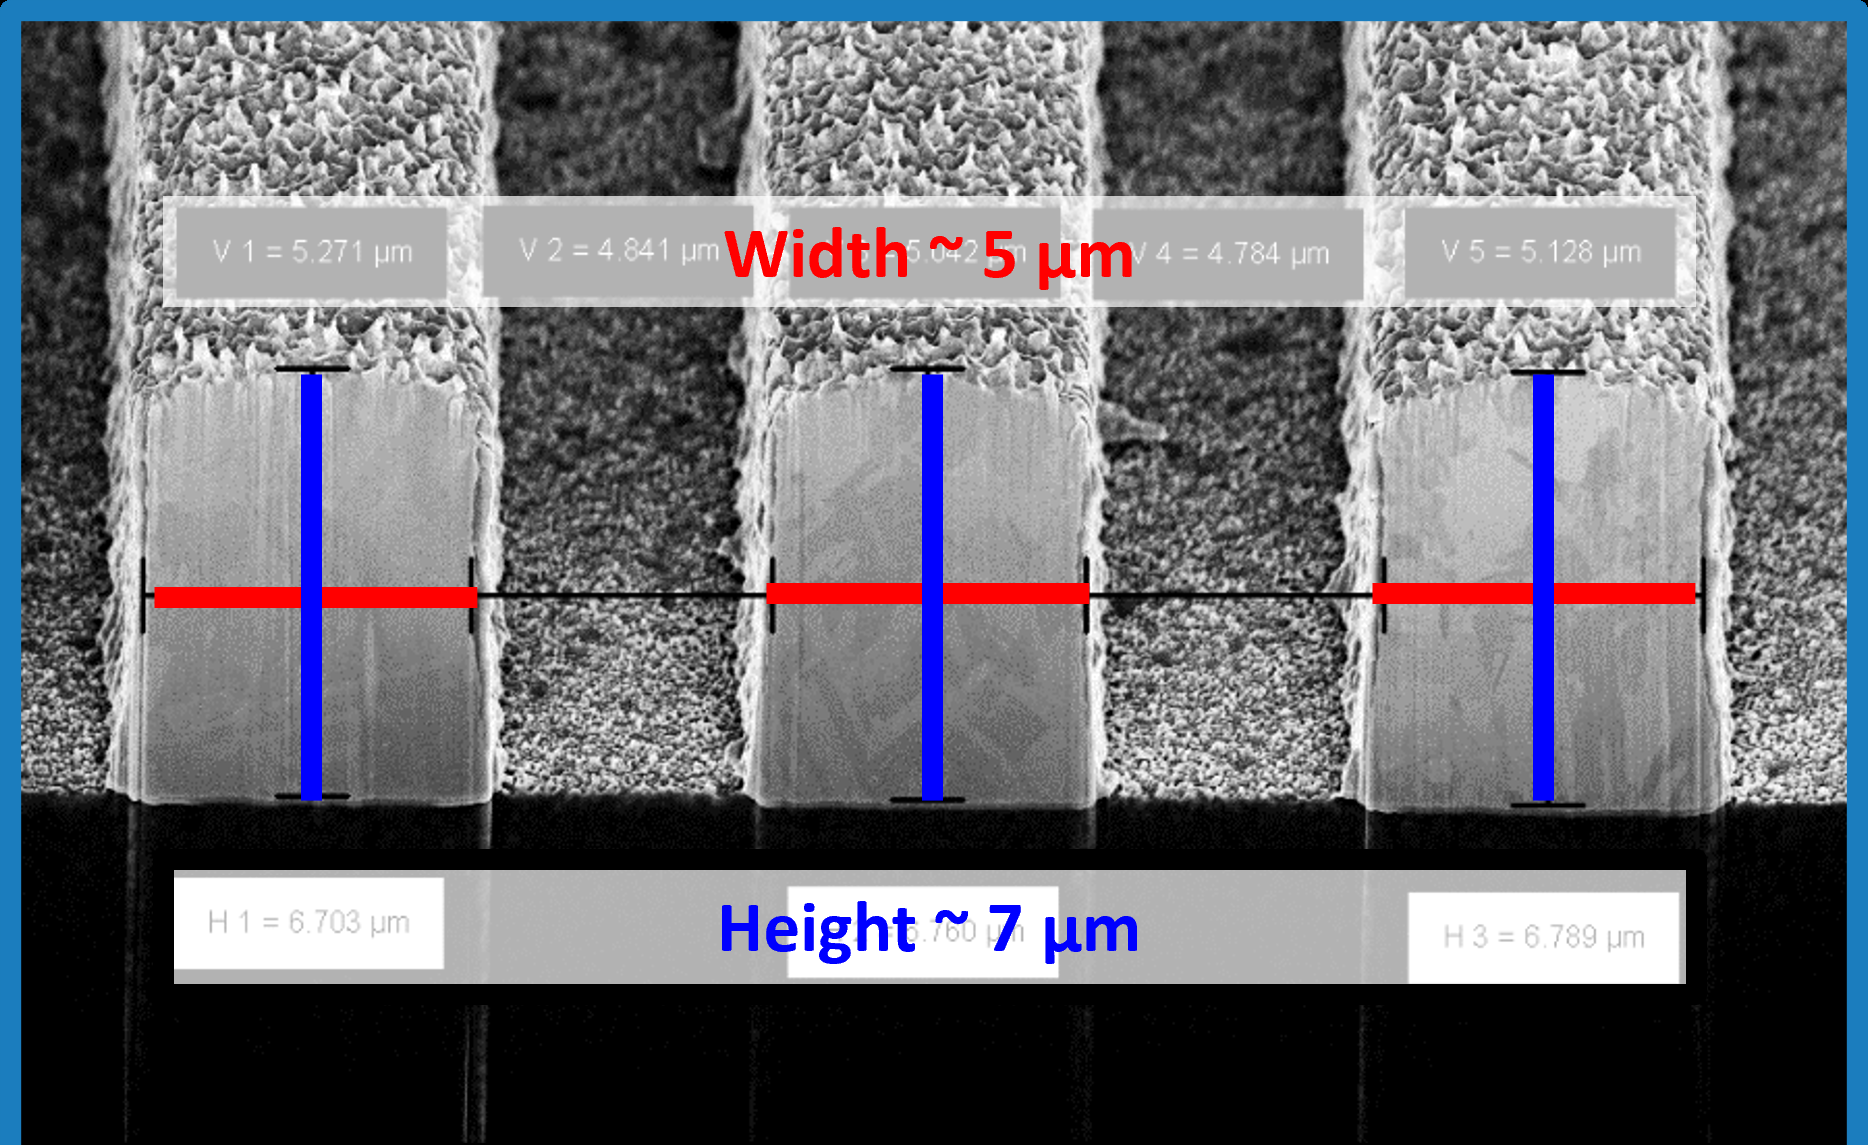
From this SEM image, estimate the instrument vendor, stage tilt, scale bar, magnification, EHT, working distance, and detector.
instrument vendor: Zeiss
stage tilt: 54°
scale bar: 2000 nm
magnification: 4 K X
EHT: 5 kV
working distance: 6.9 mm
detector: InLens